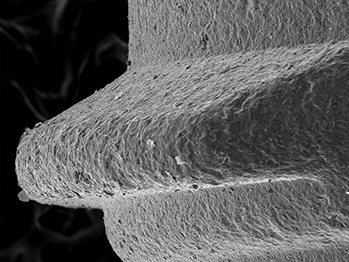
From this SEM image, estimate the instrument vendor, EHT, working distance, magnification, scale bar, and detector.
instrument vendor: other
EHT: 15 kV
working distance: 41.9 mm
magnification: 0.22 K X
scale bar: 300000 nm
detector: SE